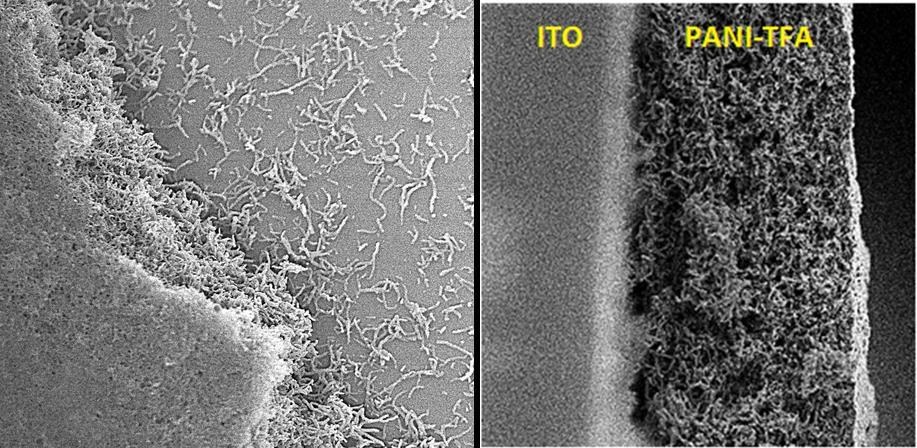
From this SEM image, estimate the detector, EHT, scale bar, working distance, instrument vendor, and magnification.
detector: SEI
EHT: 10 kV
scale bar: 10000 nm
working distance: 4.6 mm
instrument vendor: JEOL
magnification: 1.5 K X